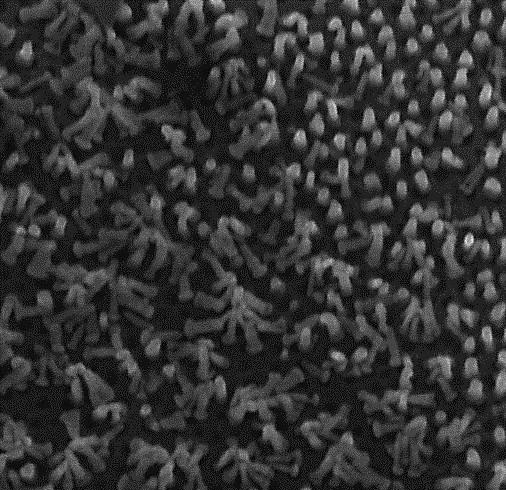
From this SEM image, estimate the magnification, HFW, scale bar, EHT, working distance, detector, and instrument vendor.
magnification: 138.05 K X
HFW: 2.093 µm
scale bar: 500 nm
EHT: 15 kV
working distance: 7.267 mm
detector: InBeam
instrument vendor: Tescan